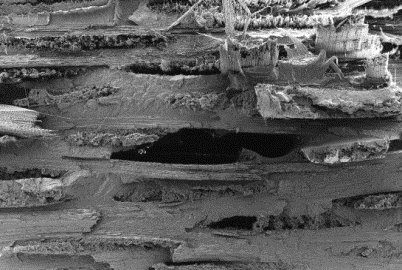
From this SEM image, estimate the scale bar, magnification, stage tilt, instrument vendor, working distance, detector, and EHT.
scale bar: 1000000 nm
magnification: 0.06 K X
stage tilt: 0°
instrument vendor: Zeiss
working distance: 6.1 mm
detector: SE2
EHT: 5 kV